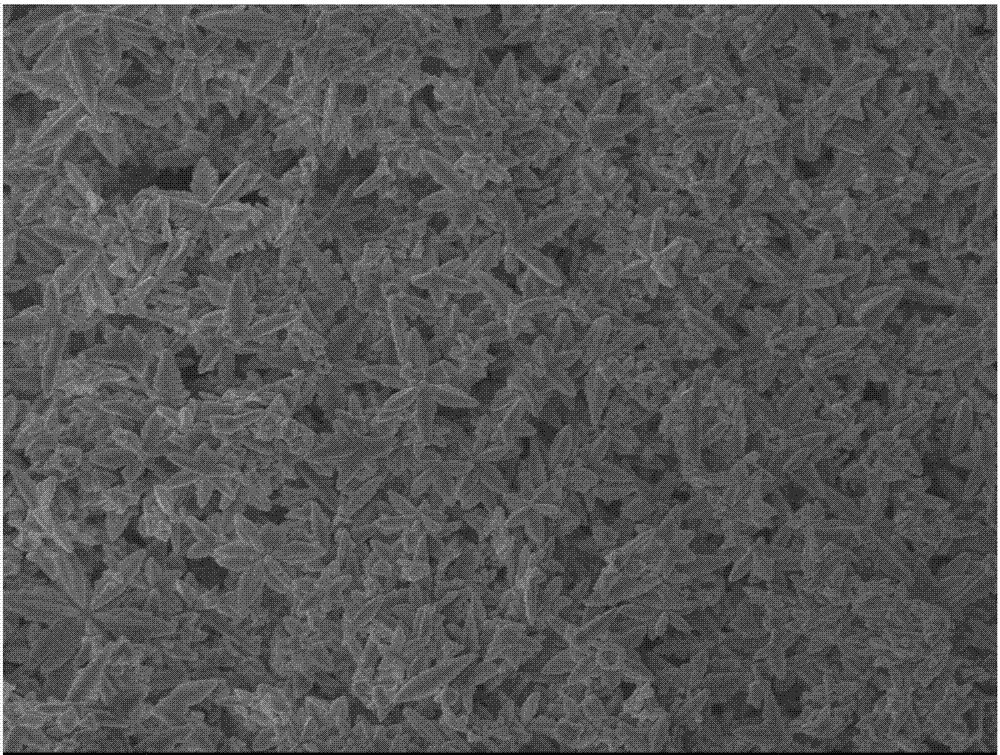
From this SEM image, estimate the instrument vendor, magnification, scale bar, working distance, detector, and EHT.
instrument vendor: JEOL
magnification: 5 K X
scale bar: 1000 nm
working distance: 7.8 mm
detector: SEI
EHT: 5 kV